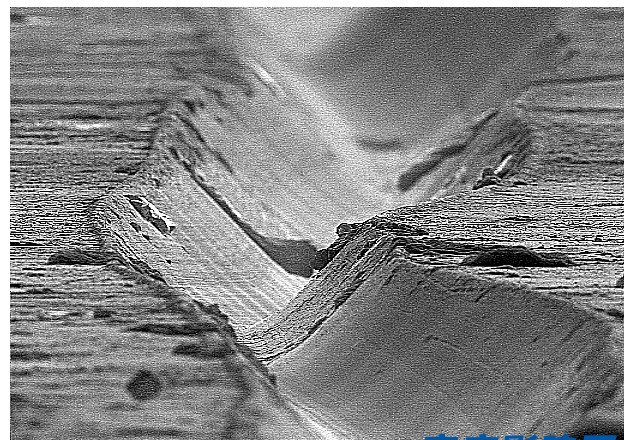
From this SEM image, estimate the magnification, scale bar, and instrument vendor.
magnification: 1 K X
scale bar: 20000 nm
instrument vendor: Hitachi